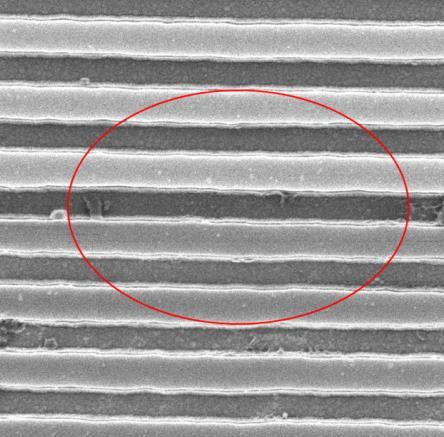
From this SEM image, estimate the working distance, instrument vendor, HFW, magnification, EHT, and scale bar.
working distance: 6.9 mm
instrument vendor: Zeiss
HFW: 2 µm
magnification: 57.15 K X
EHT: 25 kV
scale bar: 200 nm